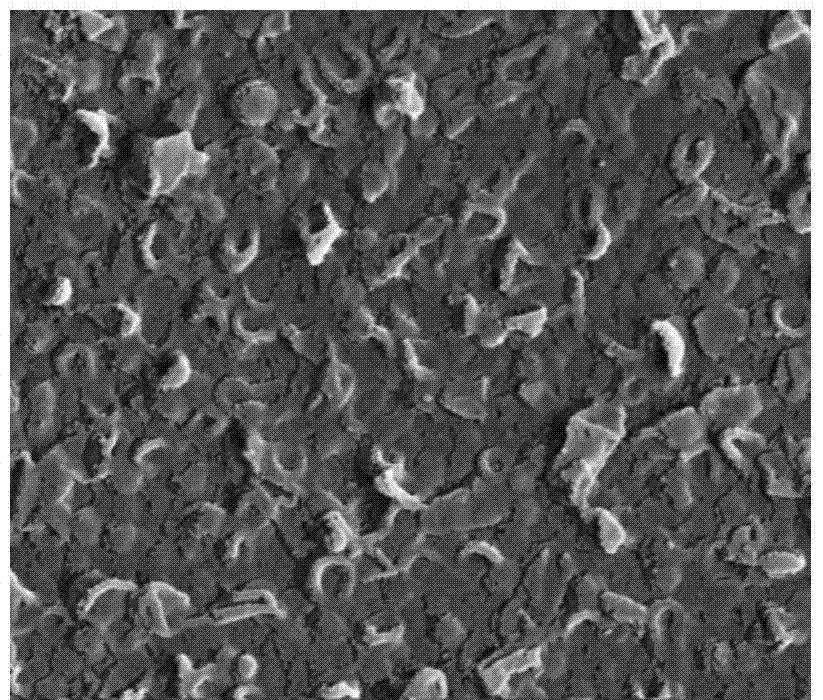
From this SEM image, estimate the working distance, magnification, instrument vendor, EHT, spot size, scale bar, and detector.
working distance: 5 mm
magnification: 80 K X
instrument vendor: FEI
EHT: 10 kV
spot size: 3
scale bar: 500 nm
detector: TLD